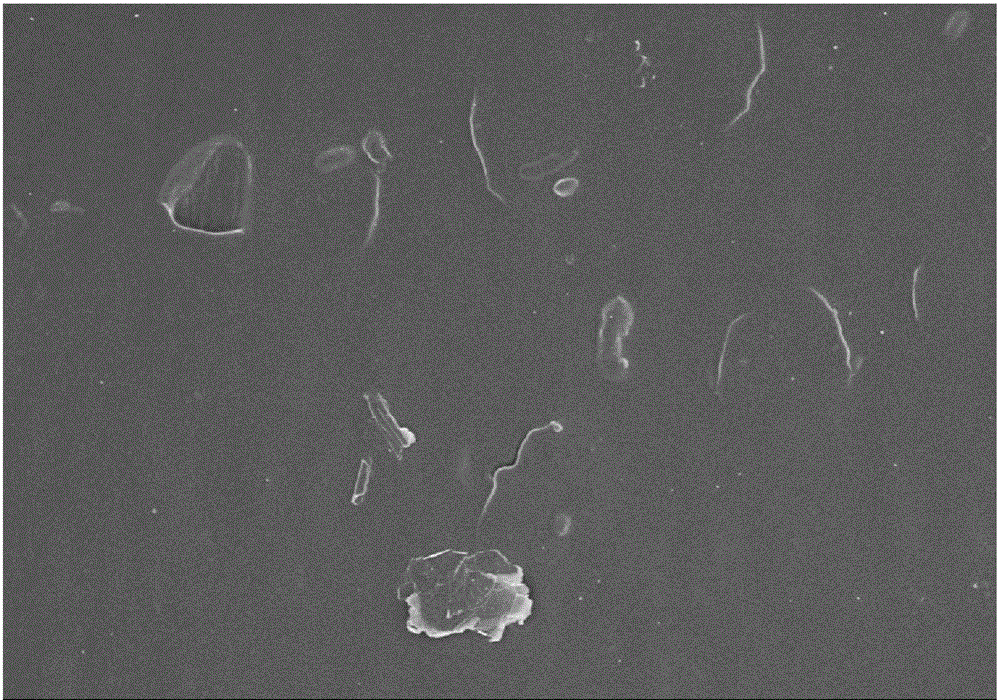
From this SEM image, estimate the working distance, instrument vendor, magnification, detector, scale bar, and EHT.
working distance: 8.8 mm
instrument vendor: Hitachi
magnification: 1 K X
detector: SE(M,LA0)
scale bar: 50000 nm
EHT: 5 kV